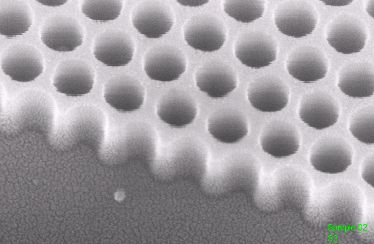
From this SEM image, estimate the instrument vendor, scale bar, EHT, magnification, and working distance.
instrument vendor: Zeiss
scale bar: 100 nm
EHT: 8 kV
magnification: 298.06 K X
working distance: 7 mm